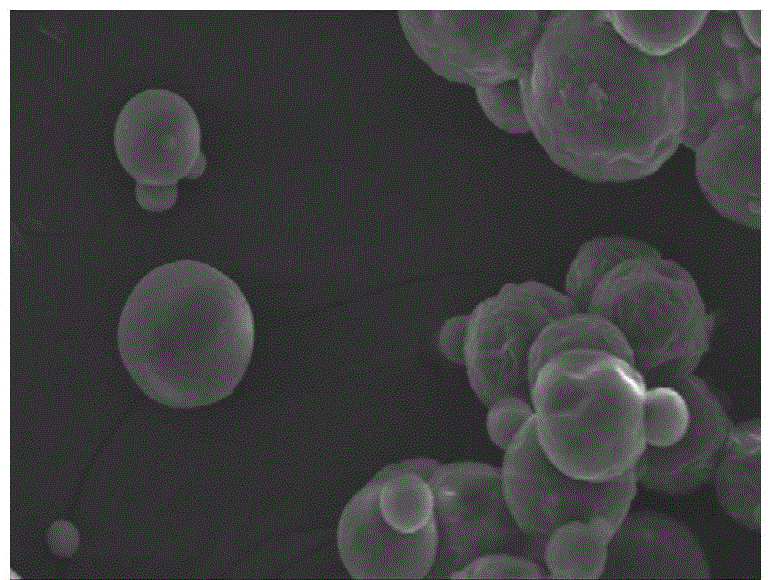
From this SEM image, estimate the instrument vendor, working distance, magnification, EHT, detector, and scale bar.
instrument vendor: Hitachi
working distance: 24.5 mm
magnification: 0.9 K X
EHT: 20 kV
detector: SE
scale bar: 50000 nm